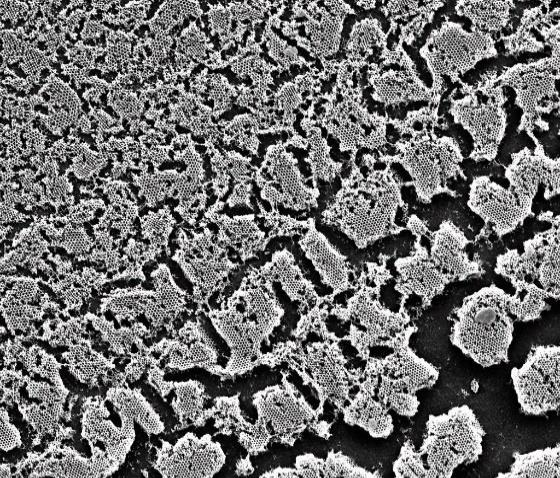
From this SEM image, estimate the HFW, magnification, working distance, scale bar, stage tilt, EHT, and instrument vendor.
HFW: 54.1 µm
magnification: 5 K X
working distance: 10.1 mm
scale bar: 20000 nm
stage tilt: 0°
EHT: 25 kV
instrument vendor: FEI